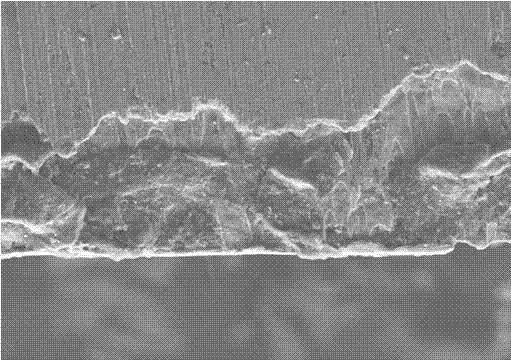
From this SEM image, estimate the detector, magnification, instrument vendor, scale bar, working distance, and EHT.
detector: LEI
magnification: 1 K X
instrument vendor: JEOL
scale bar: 10000 nm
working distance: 10 mm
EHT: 8 kV